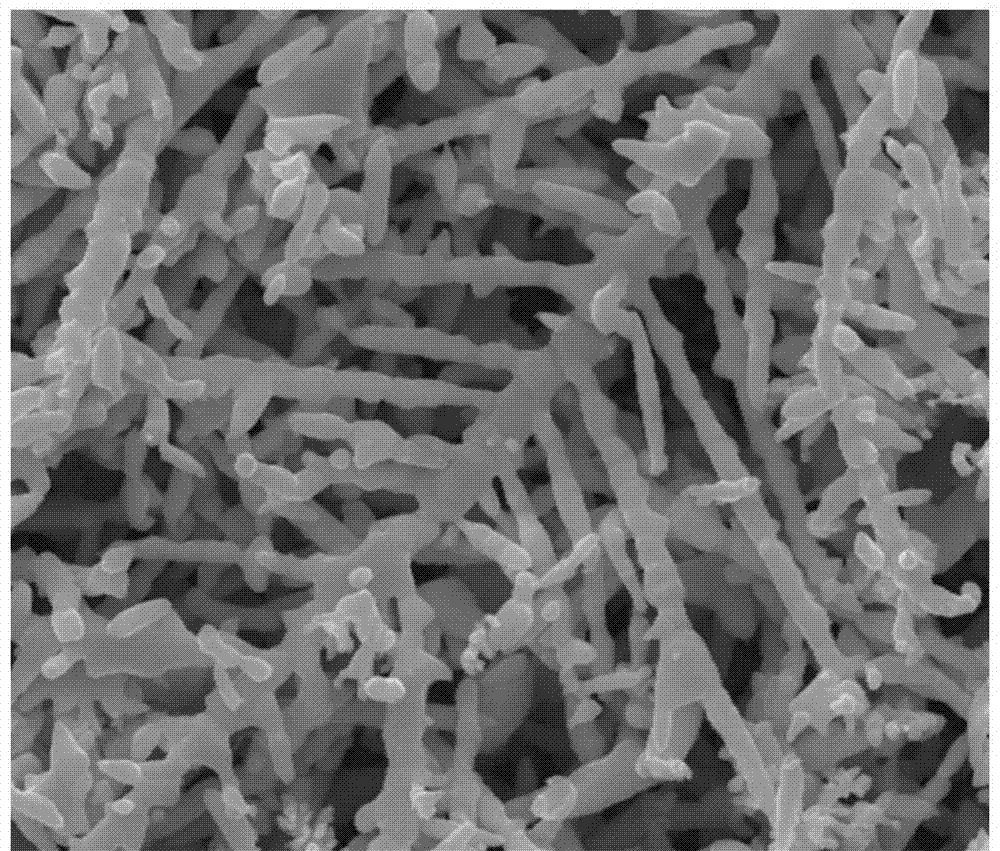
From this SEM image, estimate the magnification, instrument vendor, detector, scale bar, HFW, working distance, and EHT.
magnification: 20 K X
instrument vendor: FEI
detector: SE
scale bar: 4000 nm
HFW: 14.9 µm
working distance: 10.6 mm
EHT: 20 kV